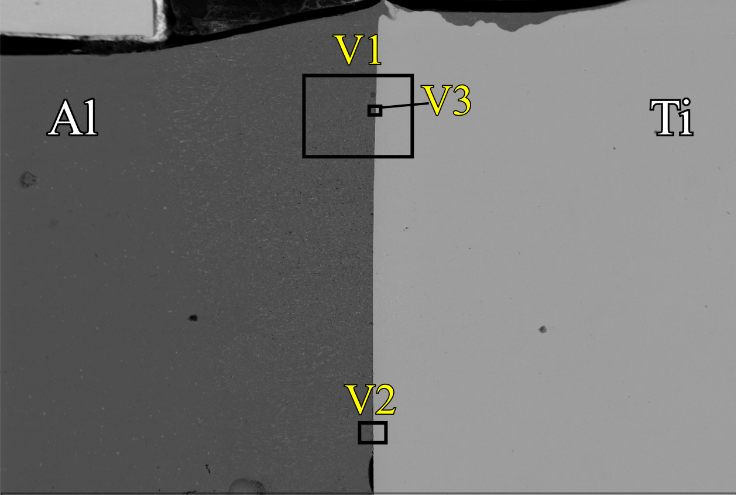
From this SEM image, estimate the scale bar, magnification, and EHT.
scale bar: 1000000 nm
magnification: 0.075 K X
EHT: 20 kV